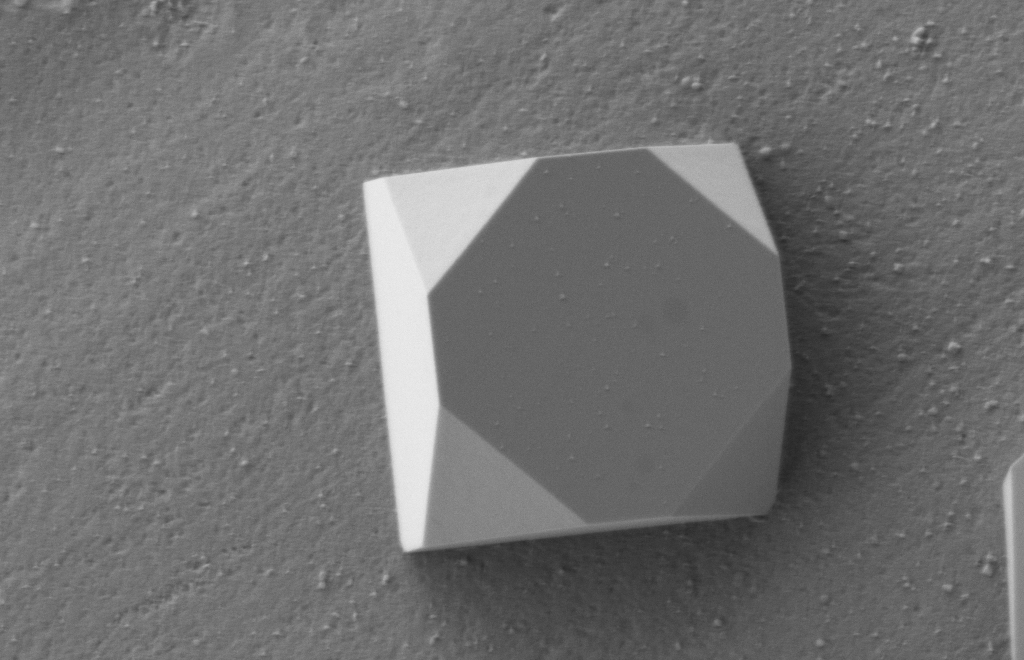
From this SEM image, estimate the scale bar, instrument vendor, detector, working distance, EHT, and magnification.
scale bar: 200 nm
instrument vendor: Zeiss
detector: SE2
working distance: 4.3 mm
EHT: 1 kV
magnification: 20 K X